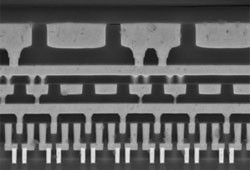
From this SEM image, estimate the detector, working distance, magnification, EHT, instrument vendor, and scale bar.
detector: SE(U,LA100)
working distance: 5.6 mm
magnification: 13 K X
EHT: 10 kV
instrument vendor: Hitachi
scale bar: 4000 nm